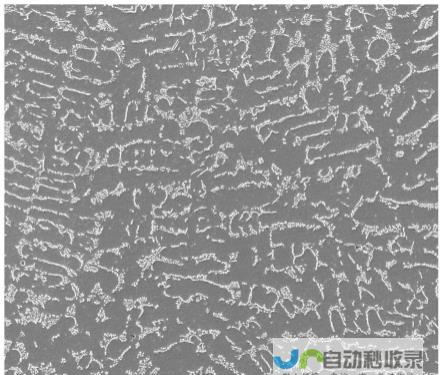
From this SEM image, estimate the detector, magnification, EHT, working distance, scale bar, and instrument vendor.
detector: ETD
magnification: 0.2 K X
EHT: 25 kV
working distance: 14.7 mm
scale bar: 300000 nm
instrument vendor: FEI